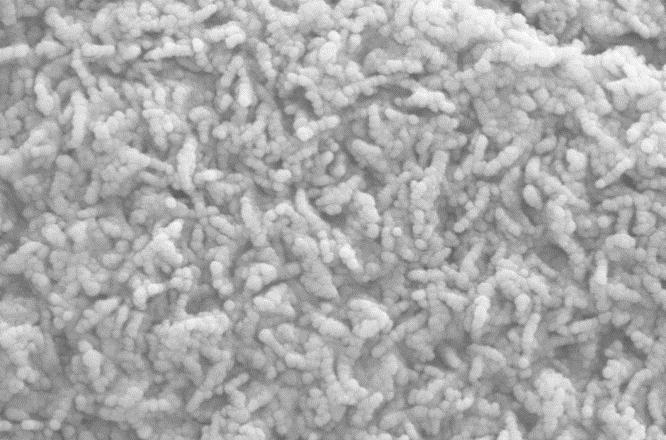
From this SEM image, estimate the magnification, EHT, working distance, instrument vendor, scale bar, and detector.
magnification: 100.71 K X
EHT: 10 kV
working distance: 7.7 mm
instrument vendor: Zeiss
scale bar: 200 nm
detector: SE2